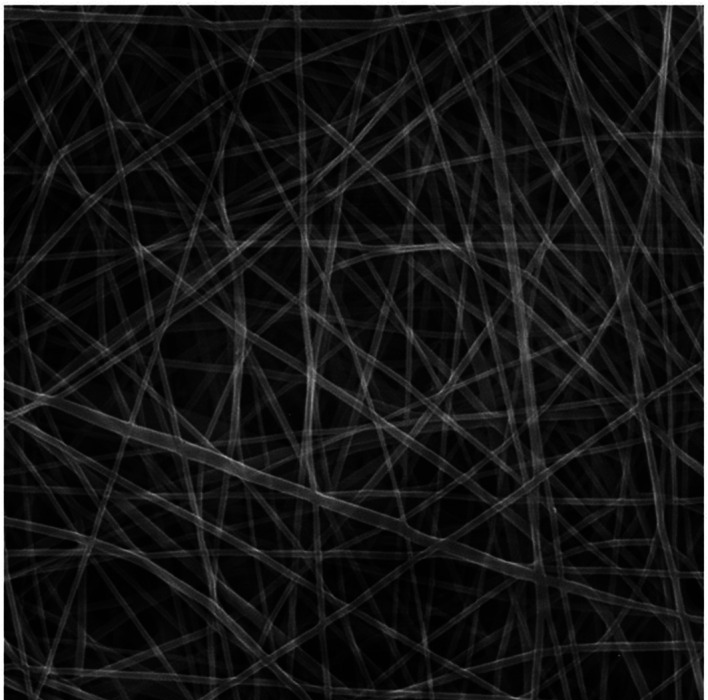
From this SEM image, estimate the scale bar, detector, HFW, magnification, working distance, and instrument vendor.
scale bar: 2000 nm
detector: SE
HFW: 12.7 µm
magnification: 15 K X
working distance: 8.21 mm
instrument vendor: Tescan